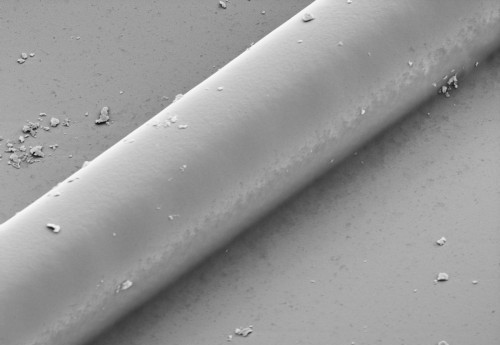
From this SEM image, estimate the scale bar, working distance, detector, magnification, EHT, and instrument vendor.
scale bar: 2000 nm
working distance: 15.6 mm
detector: SE2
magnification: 10 K X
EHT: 5 kV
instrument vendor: Zeiss